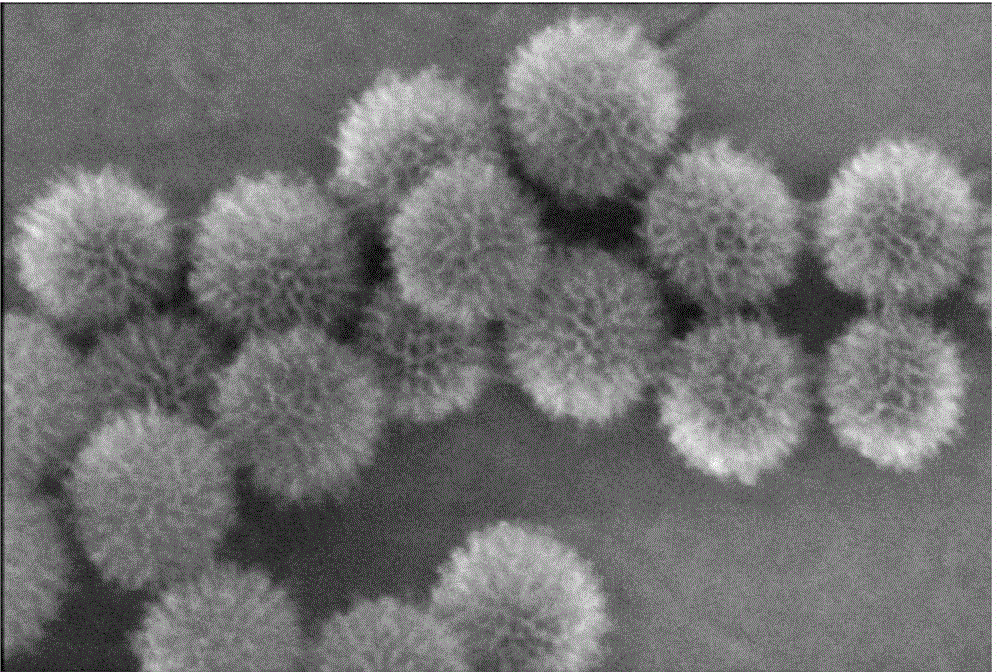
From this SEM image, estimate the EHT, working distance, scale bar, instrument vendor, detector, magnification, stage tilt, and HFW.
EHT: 10 kV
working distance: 4.1 mm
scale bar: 100 nm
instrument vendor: FEI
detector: TLD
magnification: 300 K X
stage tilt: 0°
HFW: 0.423 µm